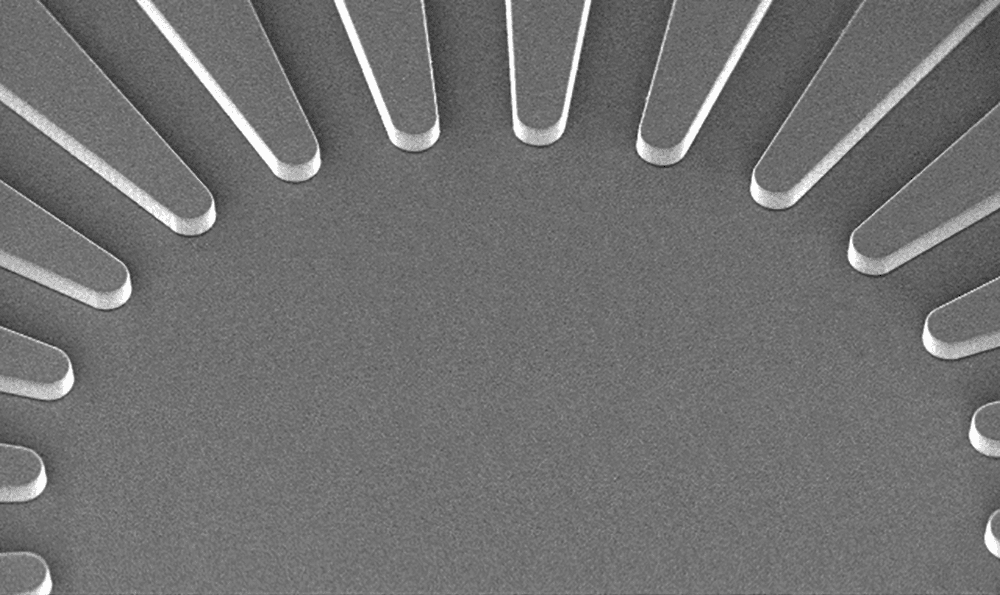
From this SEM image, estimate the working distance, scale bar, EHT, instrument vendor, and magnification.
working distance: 10.5 mm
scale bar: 500000 nm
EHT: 15 kV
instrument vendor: Hitachi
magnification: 0.06 K X